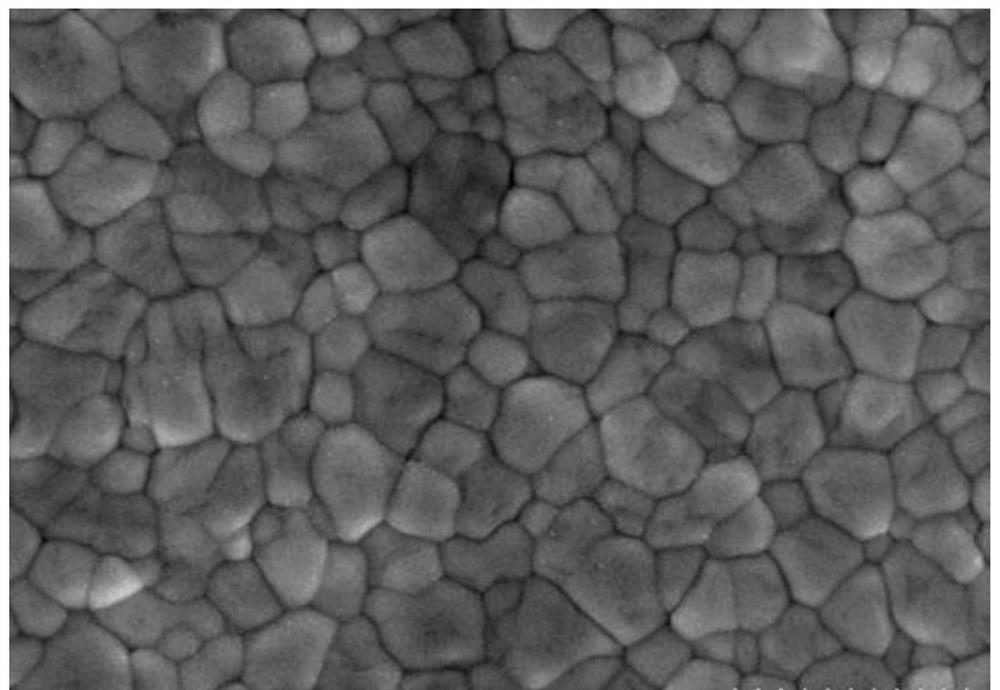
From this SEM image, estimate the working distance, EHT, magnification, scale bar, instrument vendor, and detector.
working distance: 2.9 mm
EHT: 2 kV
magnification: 60 K X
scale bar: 500 nm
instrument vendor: Hitachi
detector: SE(UL)